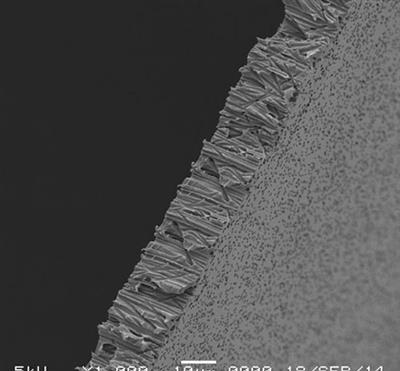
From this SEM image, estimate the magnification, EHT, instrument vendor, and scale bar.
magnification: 1 K X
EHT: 5 kV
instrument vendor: JEOL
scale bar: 10000 nm